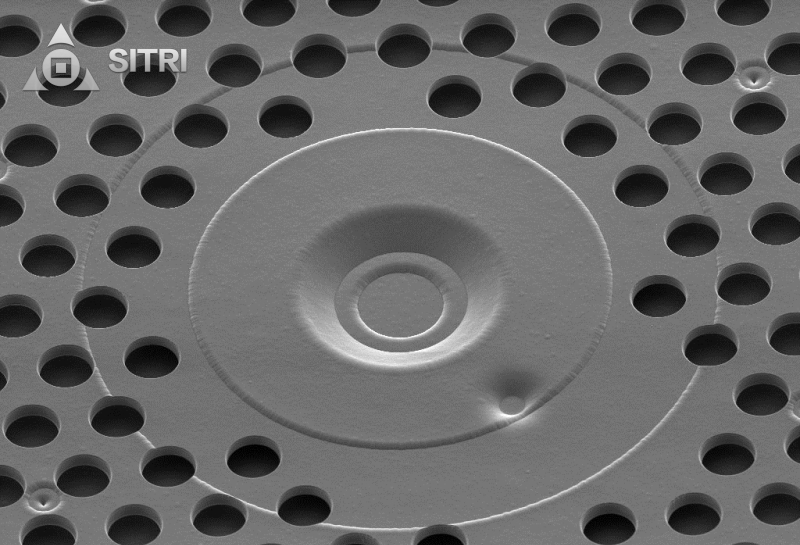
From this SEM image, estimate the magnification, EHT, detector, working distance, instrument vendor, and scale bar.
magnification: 0.75 K X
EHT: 5 kV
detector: SE2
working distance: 11.3 mm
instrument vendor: Zeiss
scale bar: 20000 nm